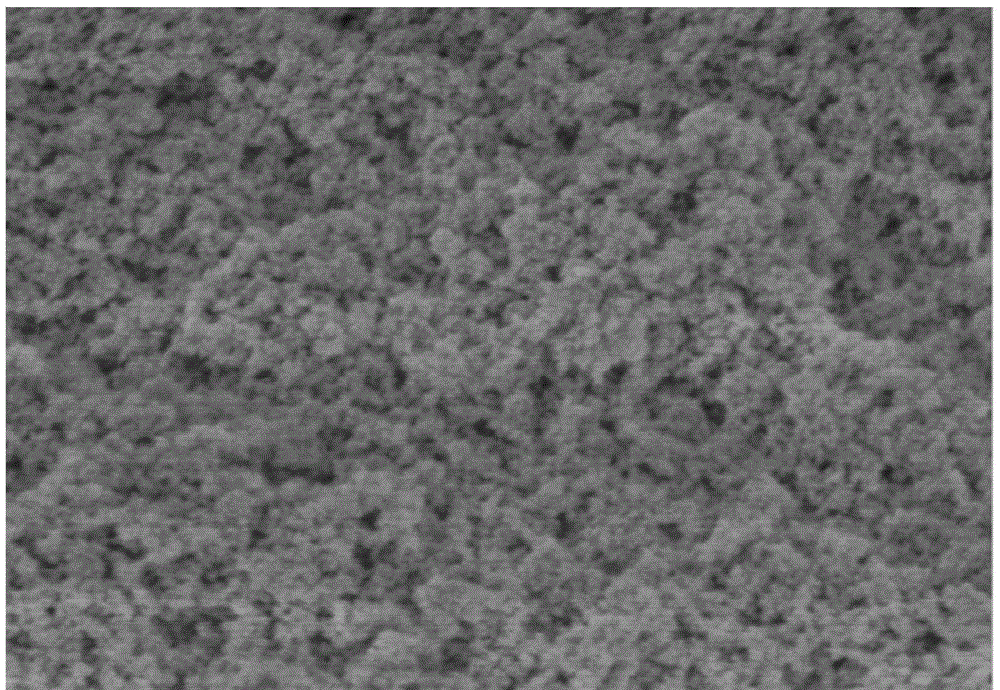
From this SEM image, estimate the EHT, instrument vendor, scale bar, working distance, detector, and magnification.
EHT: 1 kV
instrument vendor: Hitachi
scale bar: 1000 nm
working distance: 8.2 mm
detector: SE(U)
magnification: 50 K X